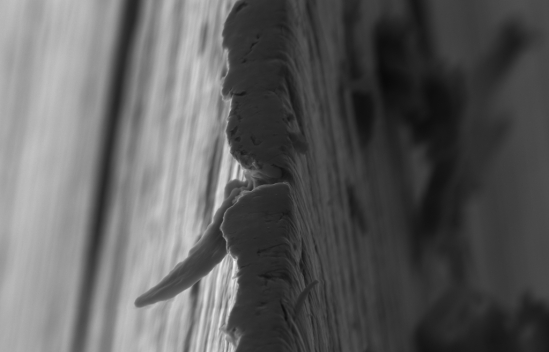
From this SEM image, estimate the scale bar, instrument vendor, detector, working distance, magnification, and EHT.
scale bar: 1000 nm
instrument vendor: Zeiss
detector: SE2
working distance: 6.5 mm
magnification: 10 K X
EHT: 5 kV